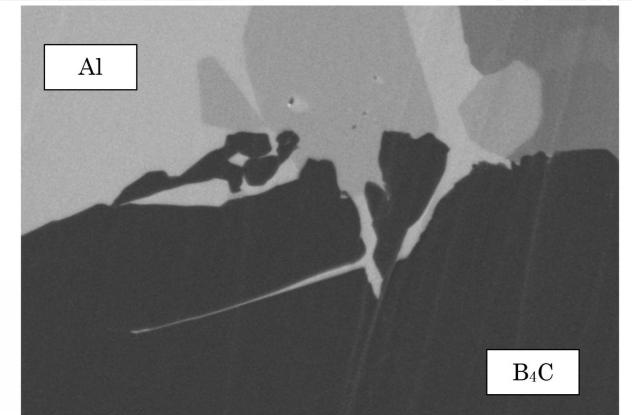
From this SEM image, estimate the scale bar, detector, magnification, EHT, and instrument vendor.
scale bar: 2000 nm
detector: SE(LA30)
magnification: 25 K X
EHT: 2 kV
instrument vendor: Hitachi